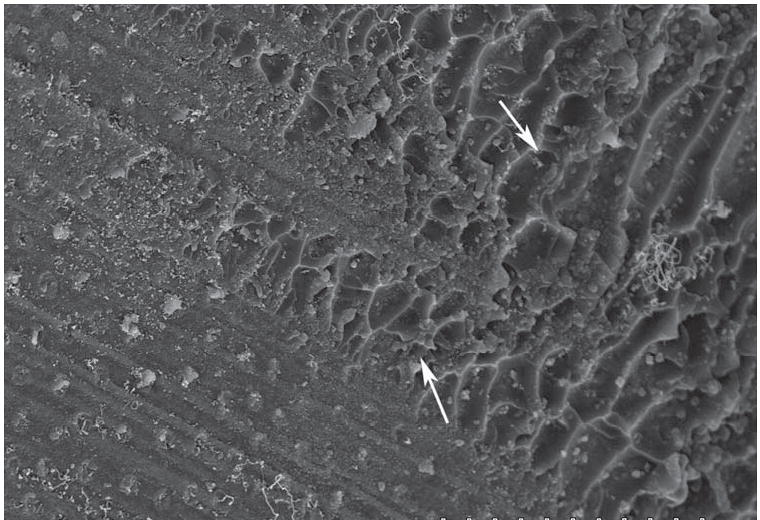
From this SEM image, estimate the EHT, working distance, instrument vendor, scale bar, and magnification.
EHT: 15 kV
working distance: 17.9 mm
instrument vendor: Hitachi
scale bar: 50000 nm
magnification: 0.9 K X